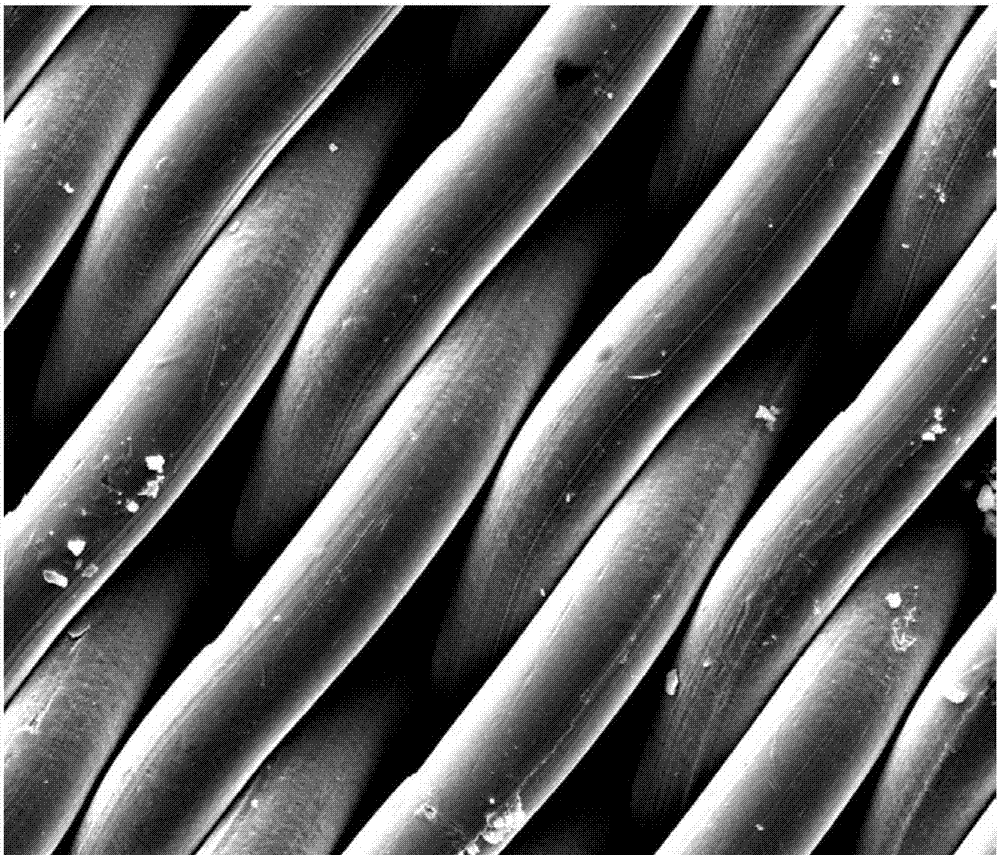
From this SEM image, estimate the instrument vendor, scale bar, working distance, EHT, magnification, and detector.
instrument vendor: FEI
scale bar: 100000 nm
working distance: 13.7 mm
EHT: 20 kV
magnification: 1.2 K X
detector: SE